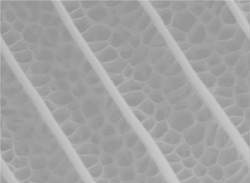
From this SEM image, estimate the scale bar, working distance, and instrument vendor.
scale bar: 5000 nm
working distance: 10.9 mm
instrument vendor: FEI